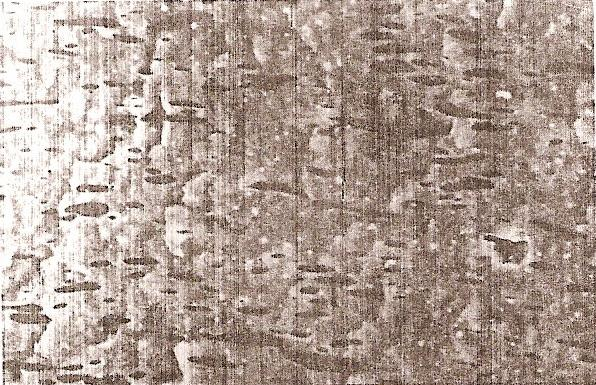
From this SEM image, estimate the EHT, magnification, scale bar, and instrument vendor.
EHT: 15 kV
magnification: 1.5 K X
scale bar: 10000 nm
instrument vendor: JEOL